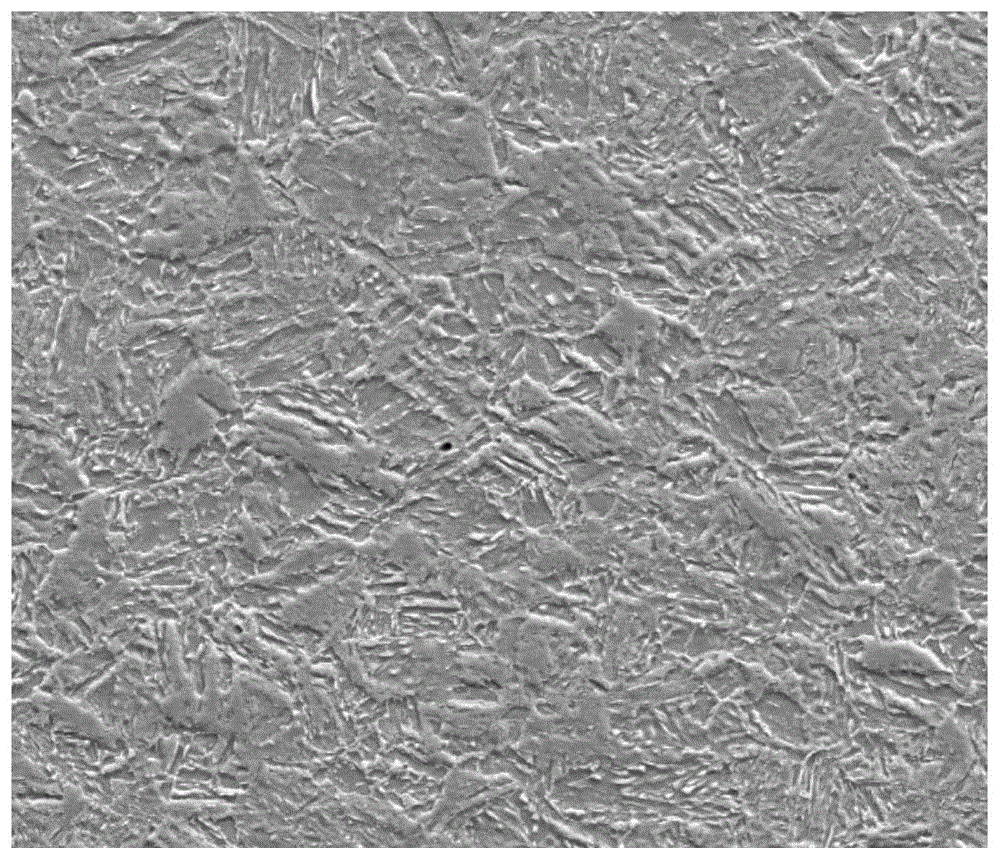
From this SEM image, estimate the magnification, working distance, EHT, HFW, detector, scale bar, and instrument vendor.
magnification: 2 K X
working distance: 18.1 mm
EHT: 25 kV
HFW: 149 µm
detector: ETD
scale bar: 50000 nm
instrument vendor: FEI